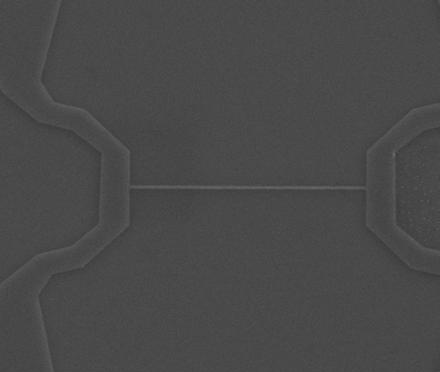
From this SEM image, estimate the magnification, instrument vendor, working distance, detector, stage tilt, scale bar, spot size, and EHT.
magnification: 30 K X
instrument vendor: FEI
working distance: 8 mm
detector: ETD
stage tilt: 0°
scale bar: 3000 nm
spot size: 2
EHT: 5 kV